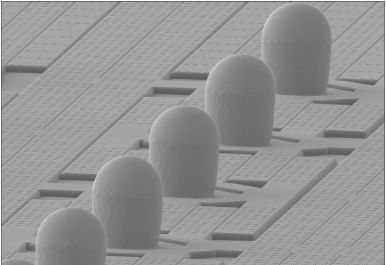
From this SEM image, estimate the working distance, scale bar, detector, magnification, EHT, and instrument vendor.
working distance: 7.5 mm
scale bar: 50000 nm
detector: SE(M)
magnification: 0.7 K X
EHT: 15 kV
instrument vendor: Hitachi